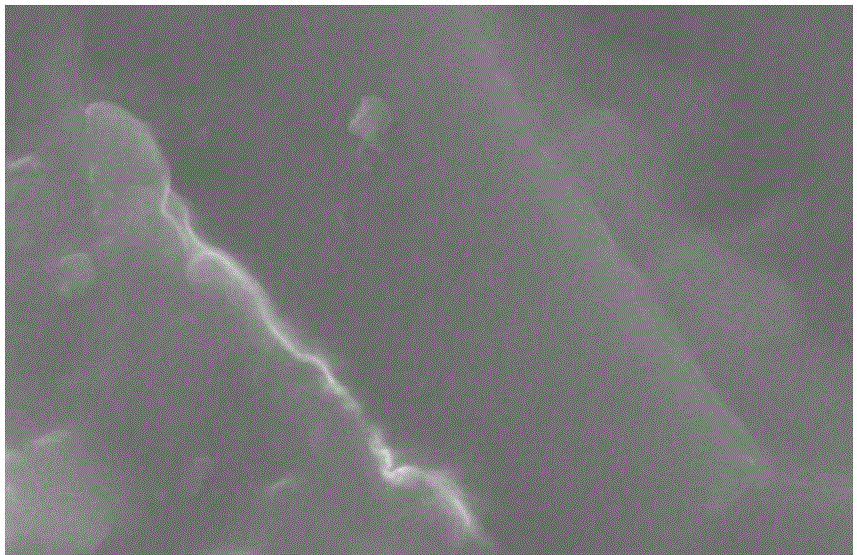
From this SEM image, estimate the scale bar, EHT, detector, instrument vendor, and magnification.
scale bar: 5000 nm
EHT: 20 kV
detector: SEI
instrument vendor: JEOL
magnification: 5 K X